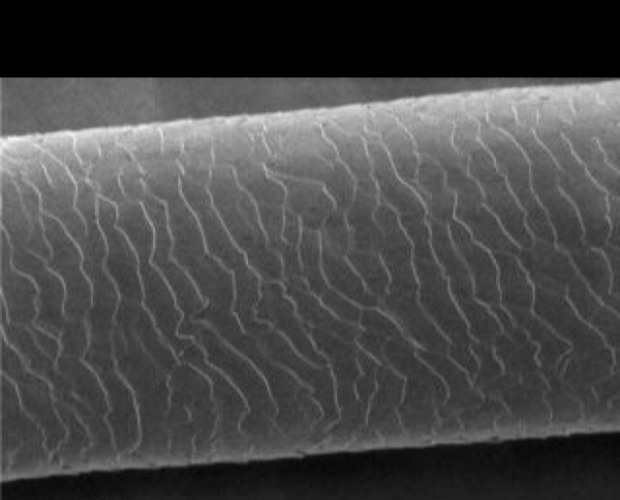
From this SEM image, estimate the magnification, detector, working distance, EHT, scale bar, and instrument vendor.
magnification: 0.7 K X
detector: S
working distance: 9.5 mm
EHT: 1 kV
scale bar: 14200 nm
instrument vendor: other